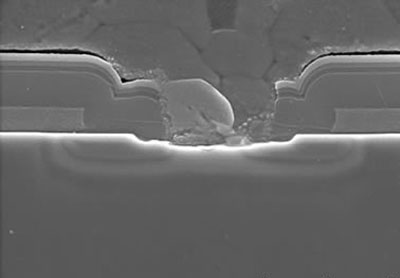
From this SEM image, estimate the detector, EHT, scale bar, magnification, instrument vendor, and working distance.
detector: SE(UL)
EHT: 5 kV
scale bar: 2000 nm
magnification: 20 K X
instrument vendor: Hitachi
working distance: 7.7 mm